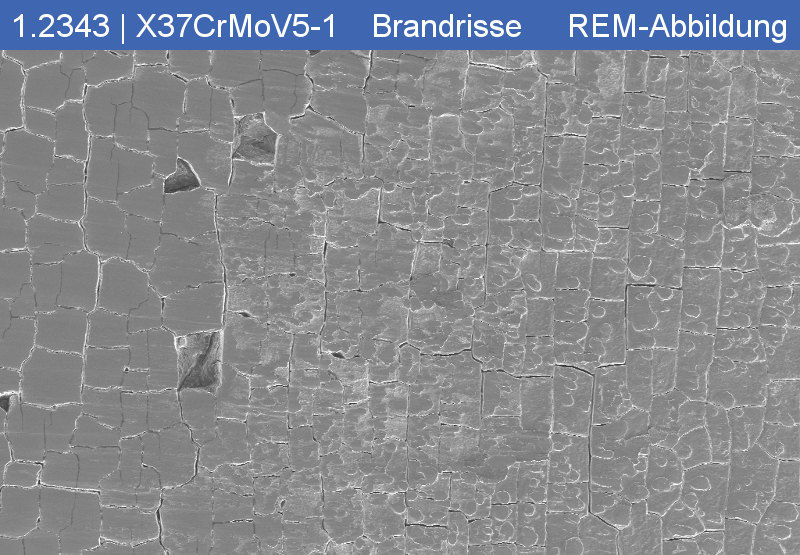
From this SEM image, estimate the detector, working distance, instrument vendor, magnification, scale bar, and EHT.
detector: SE2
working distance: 26.9 mm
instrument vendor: Zeiss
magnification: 0.02 K X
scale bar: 200000 nm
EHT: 15 kV